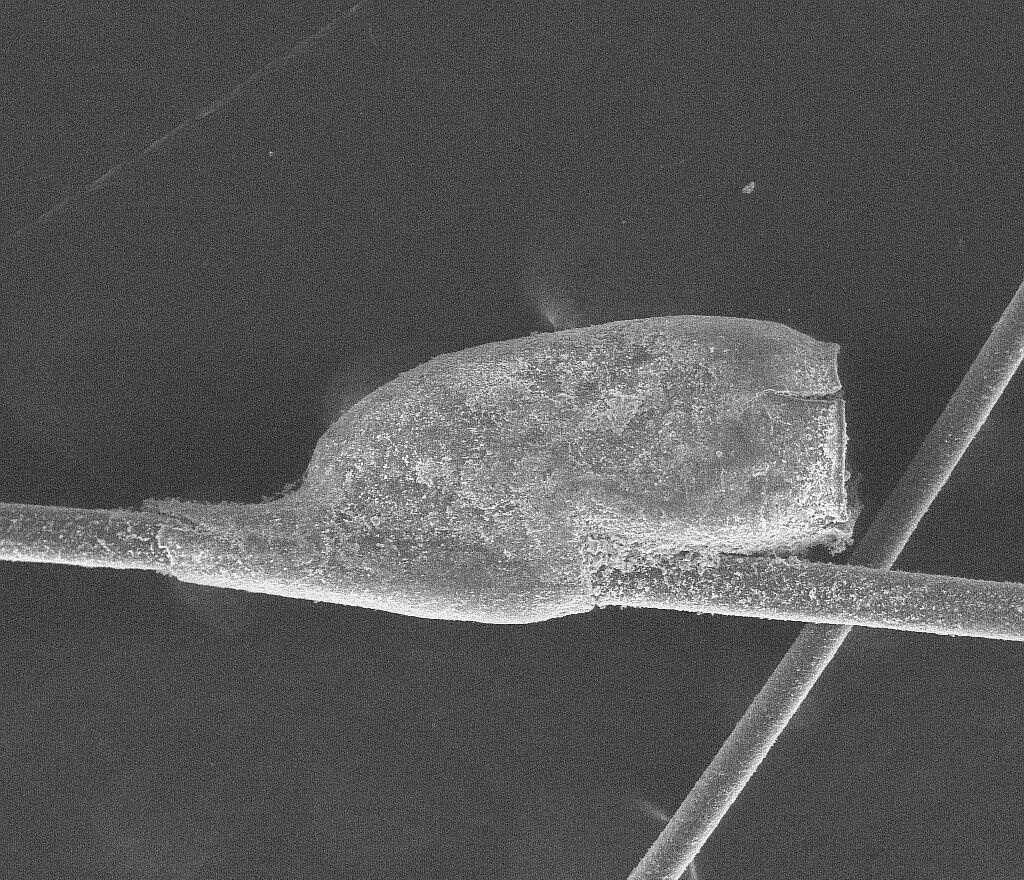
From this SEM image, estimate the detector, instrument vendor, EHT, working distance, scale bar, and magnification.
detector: LFD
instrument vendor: FEI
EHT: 15 kV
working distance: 12 mm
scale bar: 500000 nm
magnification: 0.2 K X